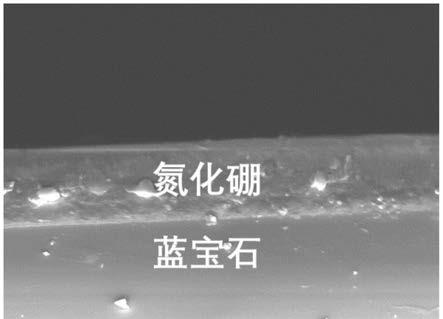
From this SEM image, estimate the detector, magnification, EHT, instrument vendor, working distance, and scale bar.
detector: SEI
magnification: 2.5 K X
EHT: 15 kV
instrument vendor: JEOL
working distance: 6.2 mm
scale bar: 10000 nm